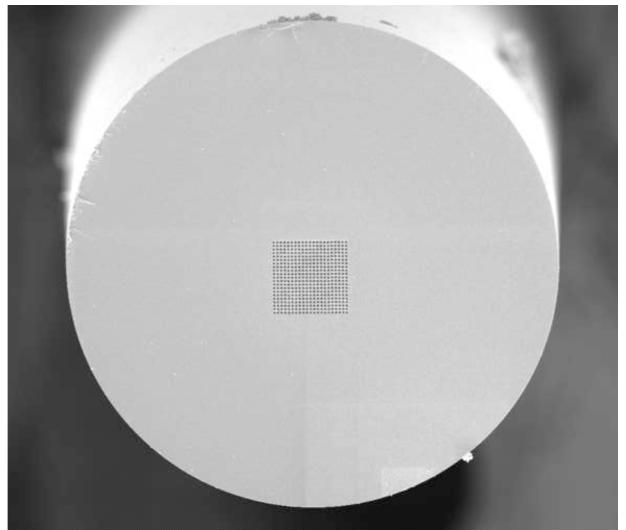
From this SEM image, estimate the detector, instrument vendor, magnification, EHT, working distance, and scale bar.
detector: ETD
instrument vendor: FEI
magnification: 0.8 K X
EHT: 5 kV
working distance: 4.1 mm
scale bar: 50000 nm